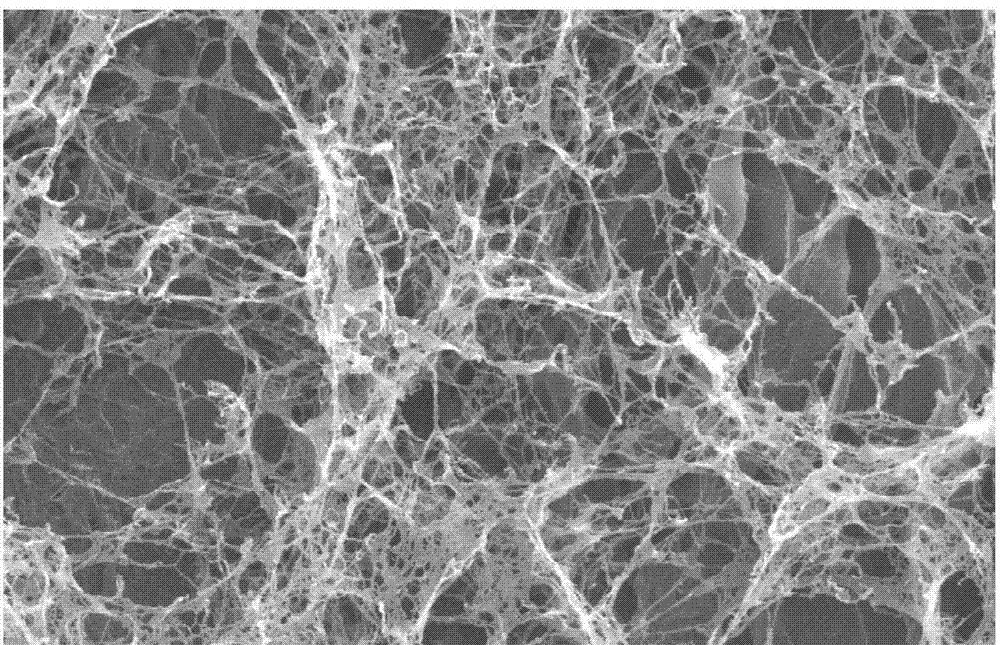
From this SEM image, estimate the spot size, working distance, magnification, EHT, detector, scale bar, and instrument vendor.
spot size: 4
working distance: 6.9 mm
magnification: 20 K X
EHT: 25 kV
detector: SE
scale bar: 2000 nm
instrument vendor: FEI